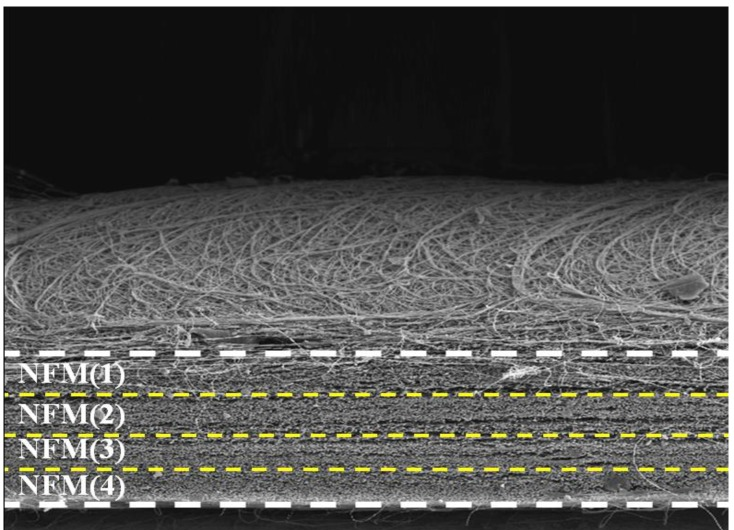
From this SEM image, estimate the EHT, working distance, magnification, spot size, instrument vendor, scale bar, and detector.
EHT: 10 kV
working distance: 4.2 mm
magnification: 0.04 K X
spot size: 3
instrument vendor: FEI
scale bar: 500000 nm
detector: SE